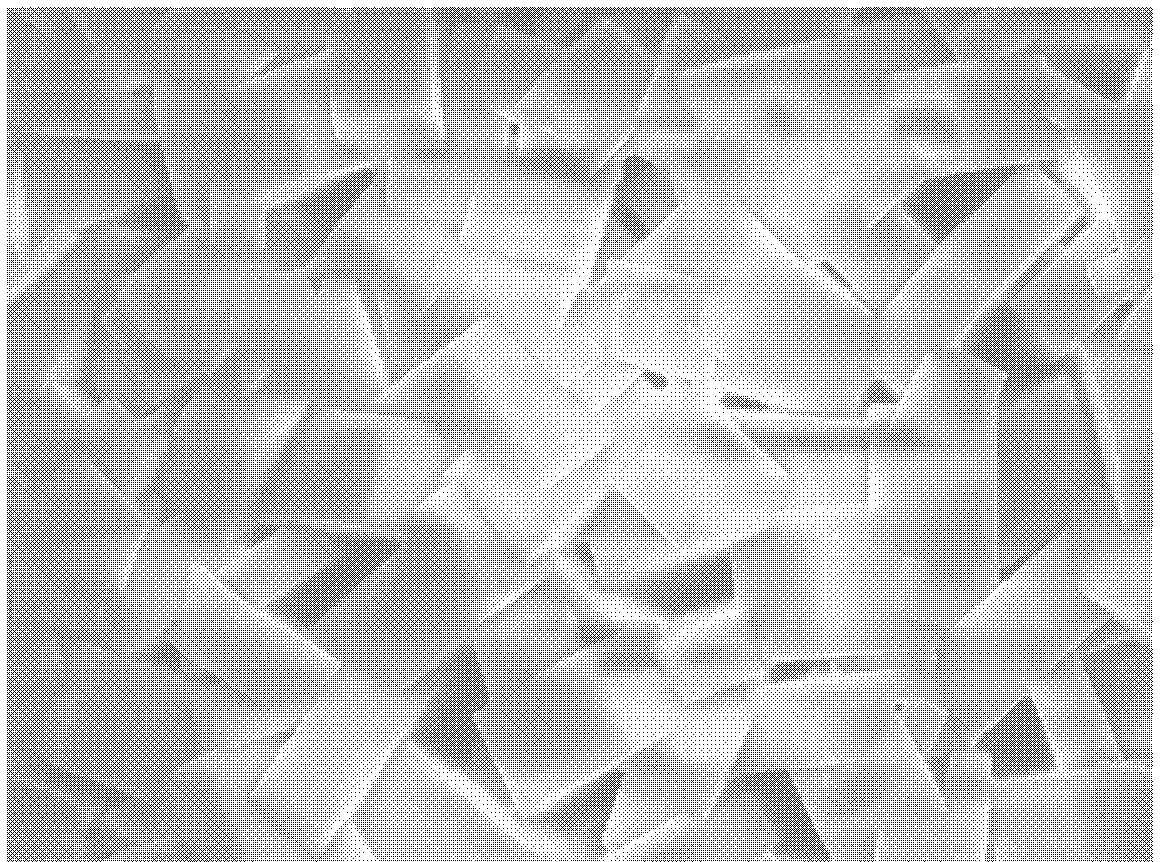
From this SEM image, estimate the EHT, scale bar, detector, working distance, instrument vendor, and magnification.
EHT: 5 kV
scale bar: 1000 nm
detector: SEI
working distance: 7.8 mm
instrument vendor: JEOL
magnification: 10 K X